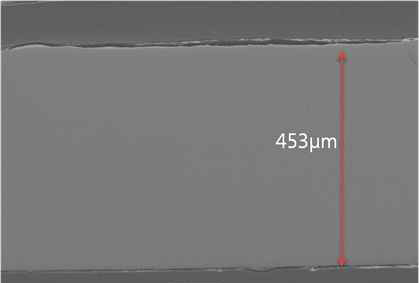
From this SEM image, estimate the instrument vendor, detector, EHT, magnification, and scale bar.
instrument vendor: JEOL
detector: SEI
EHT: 15 kV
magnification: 0.15 K X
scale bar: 100000 nm